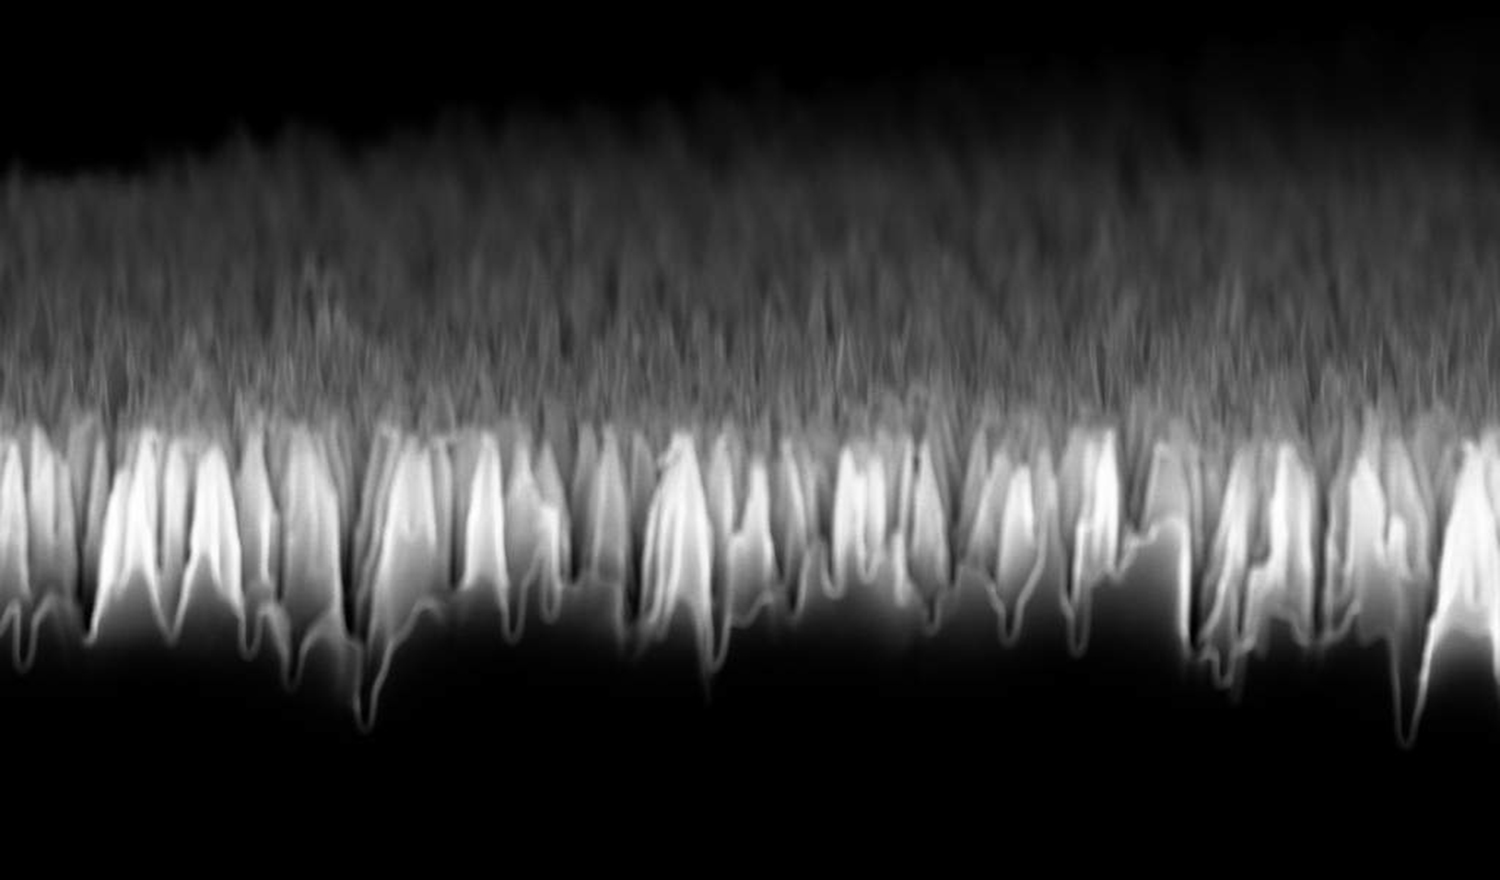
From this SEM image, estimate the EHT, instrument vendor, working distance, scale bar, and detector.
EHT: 10 kV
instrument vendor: Zeiss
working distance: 5 mm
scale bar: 1000 nm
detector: InLens